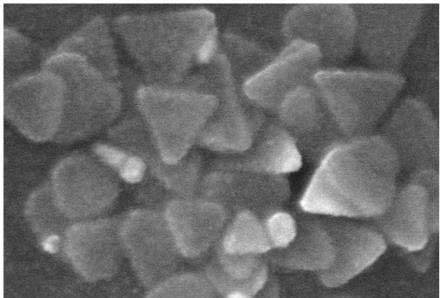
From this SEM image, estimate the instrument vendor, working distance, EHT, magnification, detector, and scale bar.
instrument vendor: Hitachi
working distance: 7.6 mm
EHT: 10 kV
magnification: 220 K X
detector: SE(TUL)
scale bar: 200 nm